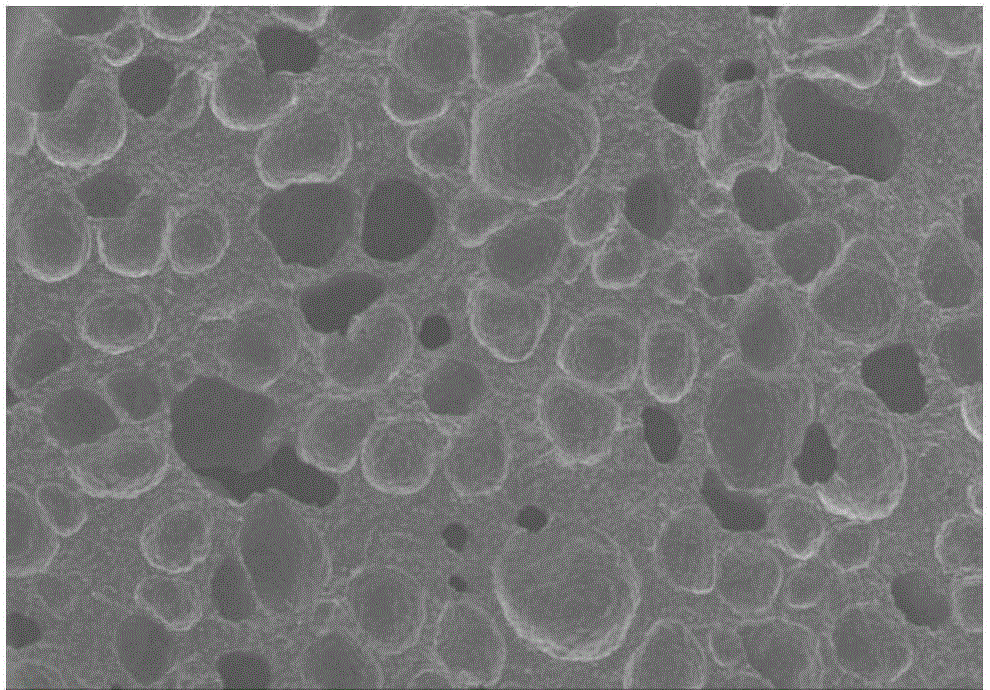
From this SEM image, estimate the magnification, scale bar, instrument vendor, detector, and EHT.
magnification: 0.06 K X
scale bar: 500000 nm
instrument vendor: other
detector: SED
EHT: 5 kV